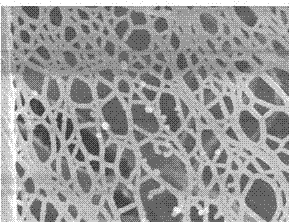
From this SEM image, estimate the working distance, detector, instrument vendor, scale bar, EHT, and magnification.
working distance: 5.3 mm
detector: SEI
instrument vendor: JEOL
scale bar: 100 nm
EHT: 5 kV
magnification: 50 K X